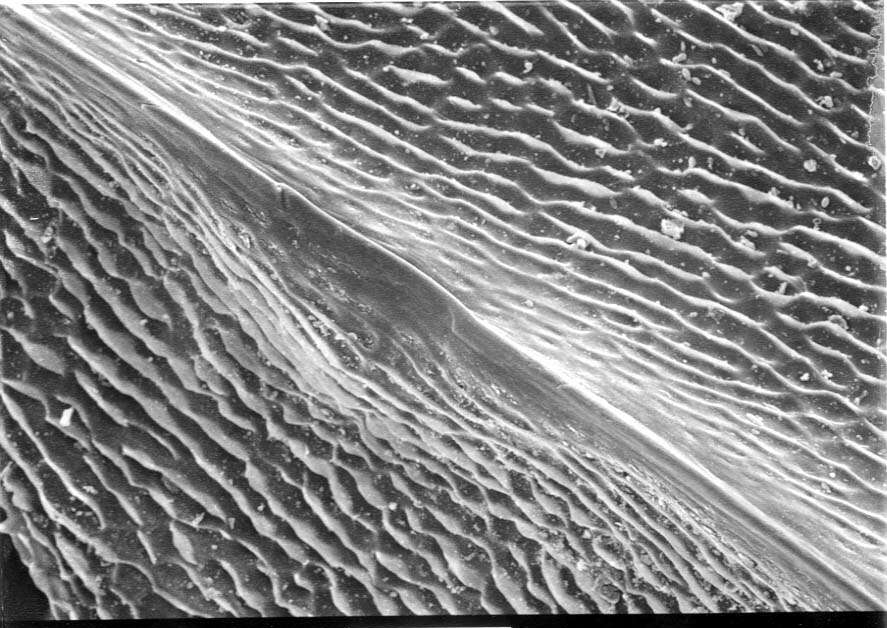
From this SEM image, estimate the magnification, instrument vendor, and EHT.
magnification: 0.2 K X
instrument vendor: JEOL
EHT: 20 kV